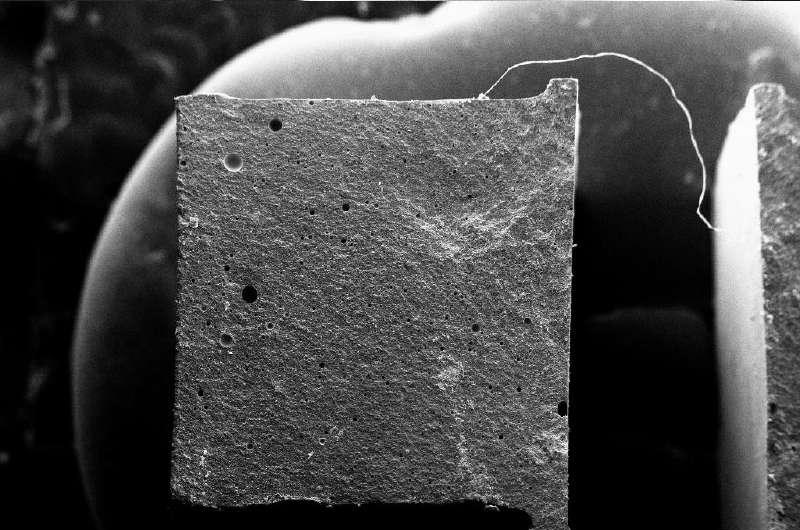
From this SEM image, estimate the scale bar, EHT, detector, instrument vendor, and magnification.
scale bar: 1000000 nm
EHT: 20 kV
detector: SE1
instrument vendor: Zeiss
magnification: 0.085 K X